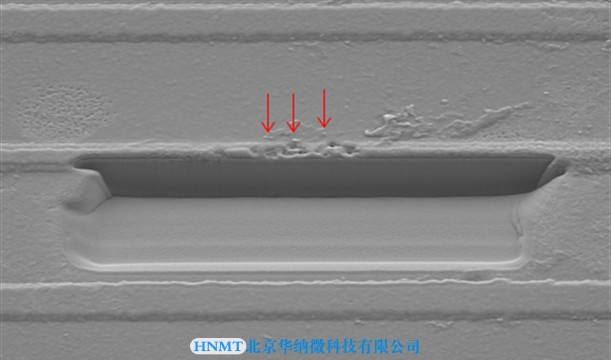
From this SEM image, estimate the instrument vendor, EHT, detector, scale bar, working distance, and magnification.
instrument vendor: Hitachi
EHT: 5 kV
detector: SE(M)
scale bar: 10000 nm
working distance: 18.1 mm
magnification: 3 K X